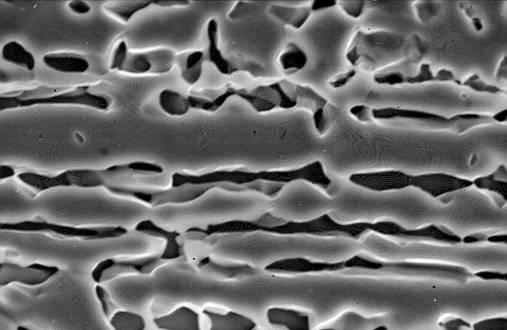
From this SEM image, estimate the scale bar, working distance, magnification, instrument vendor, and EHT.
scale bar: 10000 nm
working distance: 38 mm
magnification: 3.5 K X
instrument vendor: JEOL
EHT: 20 kV